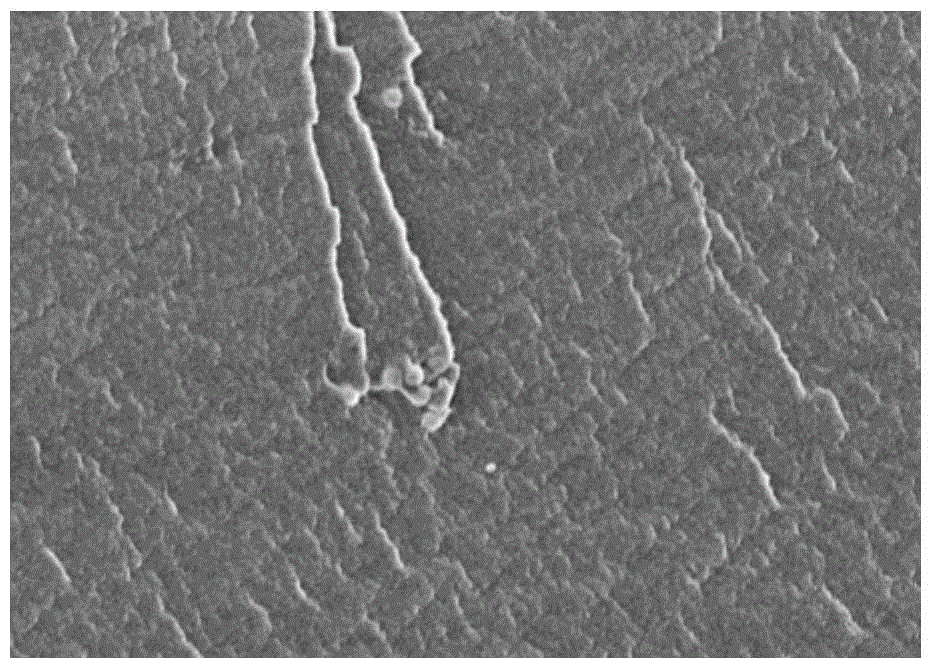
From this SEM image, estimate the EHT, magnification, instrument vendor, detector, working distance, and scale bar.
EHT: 3 kV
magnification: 10 K X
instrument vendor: Hitachi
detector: SE(M)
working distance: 8.9 mm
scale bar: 5000 nm